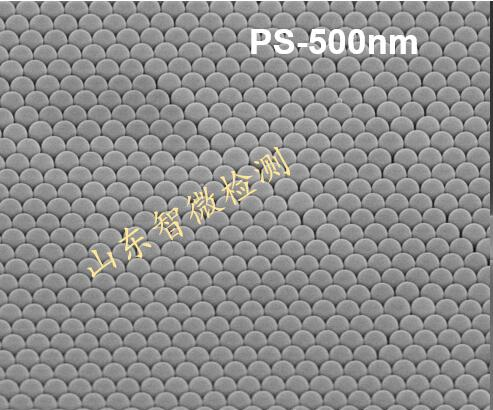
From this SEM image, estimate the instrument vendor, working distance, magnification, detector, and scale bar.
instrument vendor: Hitachi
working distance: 12.9 mm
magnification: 10 K X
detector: SE(TU)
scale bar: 5000 nm